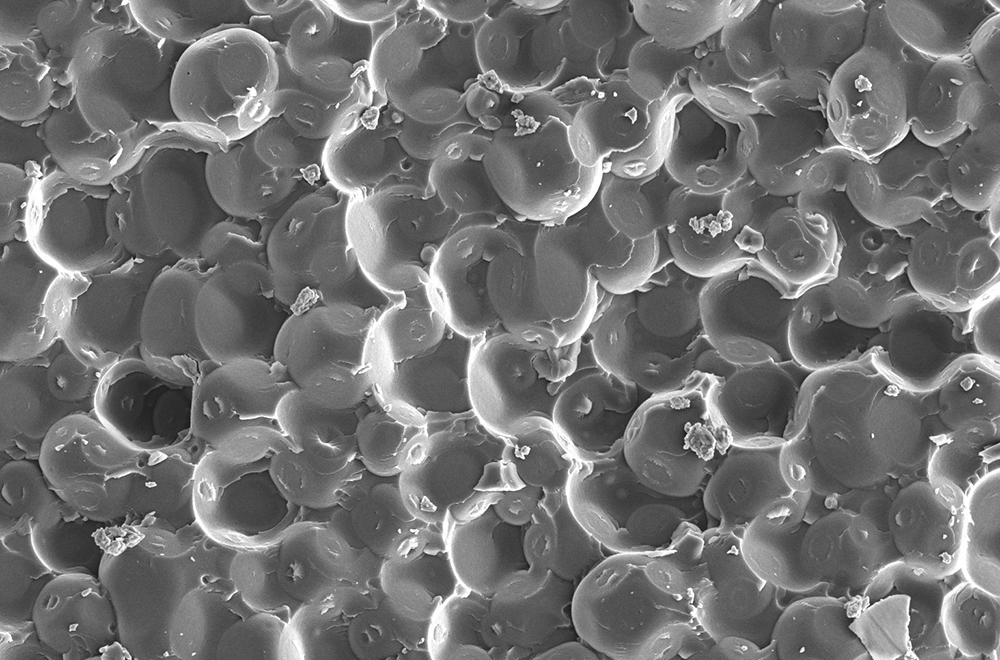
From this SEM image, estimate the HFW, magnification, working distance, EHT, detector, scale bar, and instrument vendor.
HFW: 79.4 µm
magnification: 1.6 K X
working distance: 9.2 mm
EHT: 5 kV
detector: ETD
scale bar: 30000 nm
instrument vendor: FEI